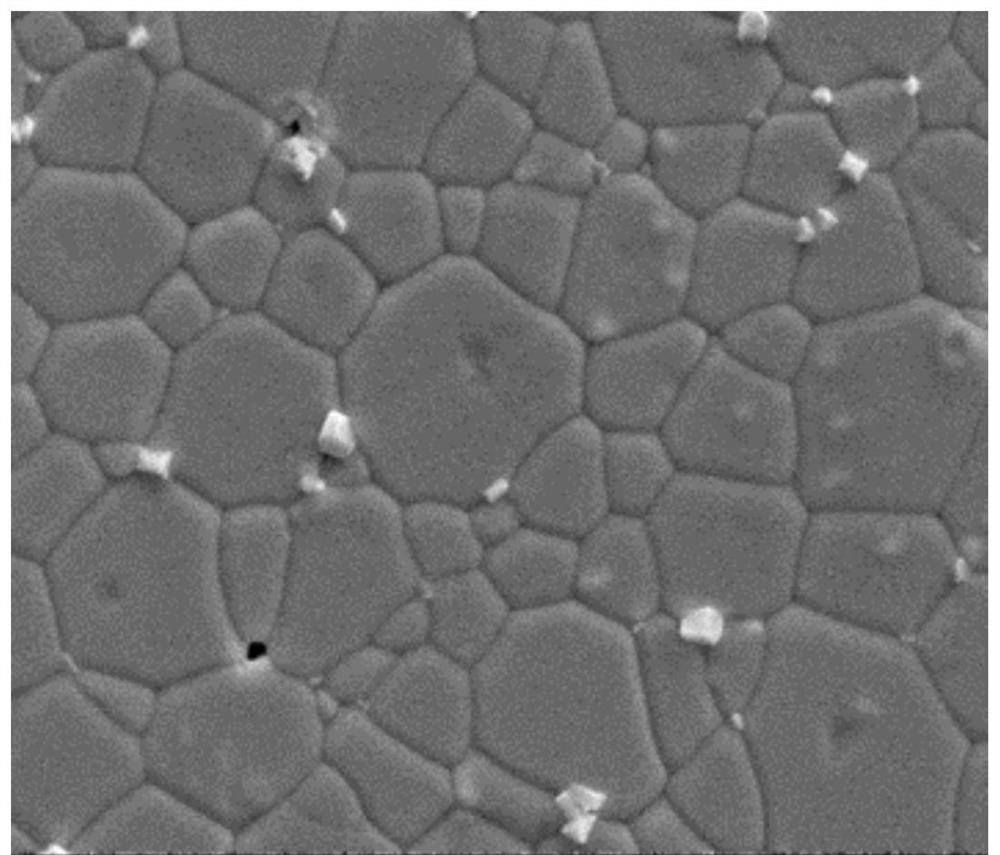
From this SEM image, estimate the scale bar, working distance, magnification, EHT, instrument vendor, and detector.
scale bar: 4000 nm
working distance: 5.8 mm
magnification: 20 K X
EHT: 10 kV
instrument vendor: FEI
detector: ETD